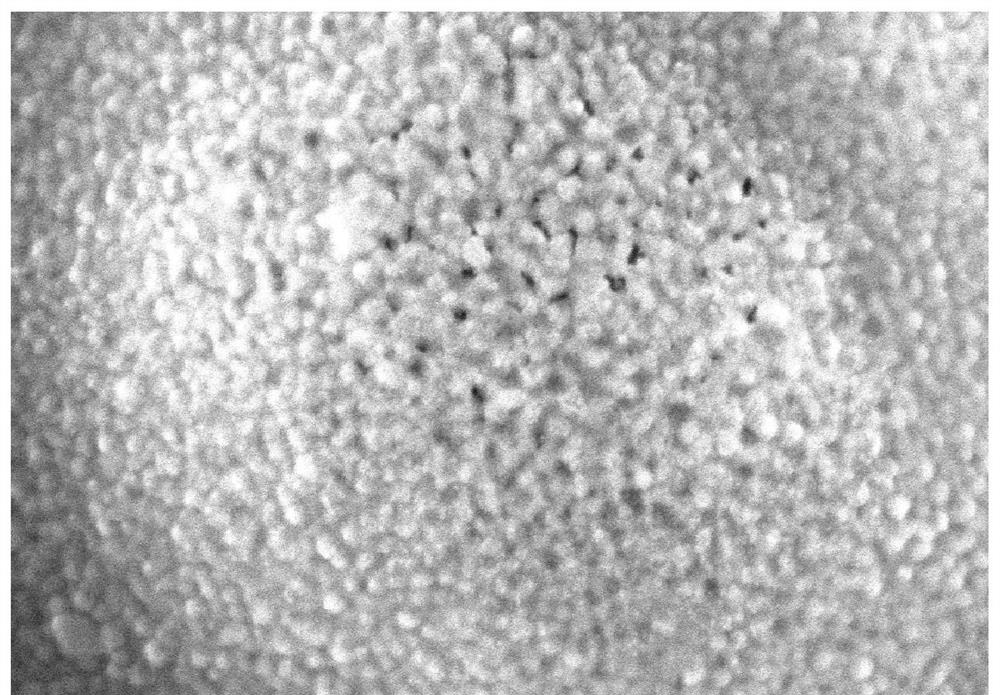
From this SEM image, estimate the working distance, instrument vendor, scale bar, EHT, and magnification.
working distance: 6 mm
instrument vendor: JEOL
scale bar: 200 nm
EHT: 10 kV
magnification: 100 K X